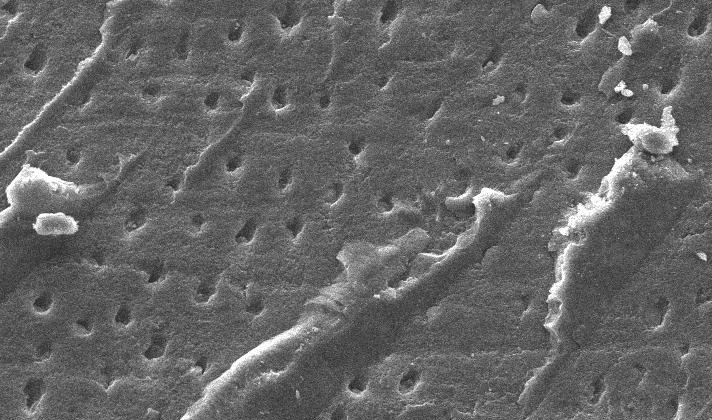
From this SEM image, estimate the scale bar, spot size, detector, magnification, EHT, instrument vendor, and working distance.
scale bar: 20000 nm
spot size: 4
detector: SE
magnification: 0.8 K X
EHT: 10 kV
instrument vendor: FEI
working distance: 10.8 mm